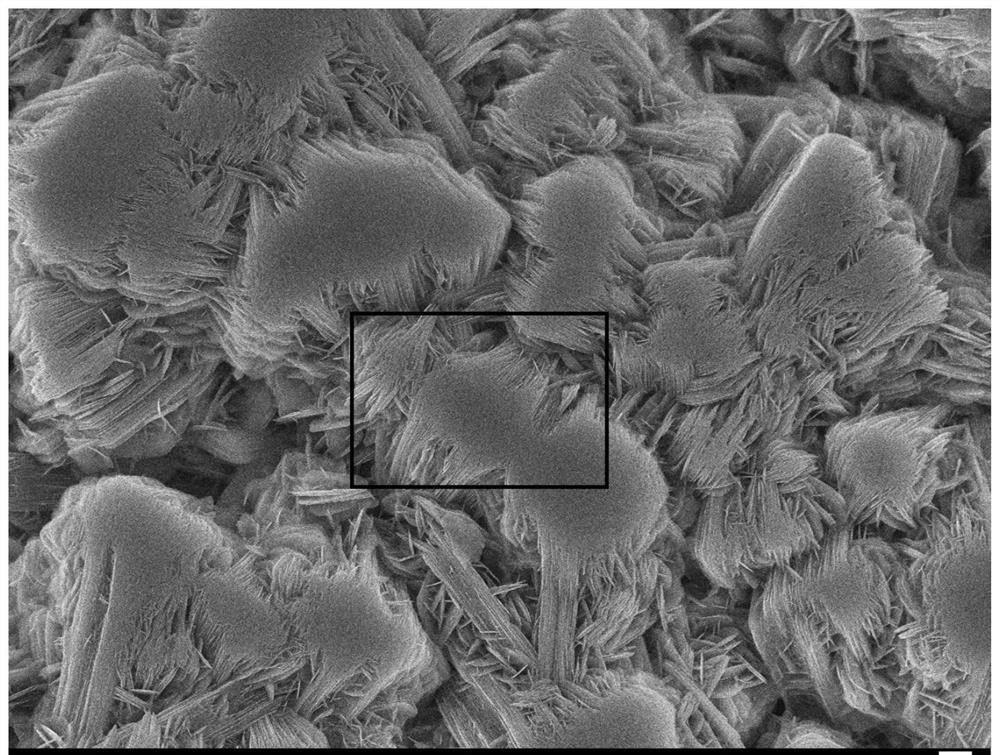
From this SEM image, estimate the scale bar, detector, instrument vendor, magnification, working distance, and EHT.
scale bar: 1000 nm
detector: SEI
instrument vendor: JEOL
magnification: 4 K X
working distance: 10.7 mm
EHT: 15 kV